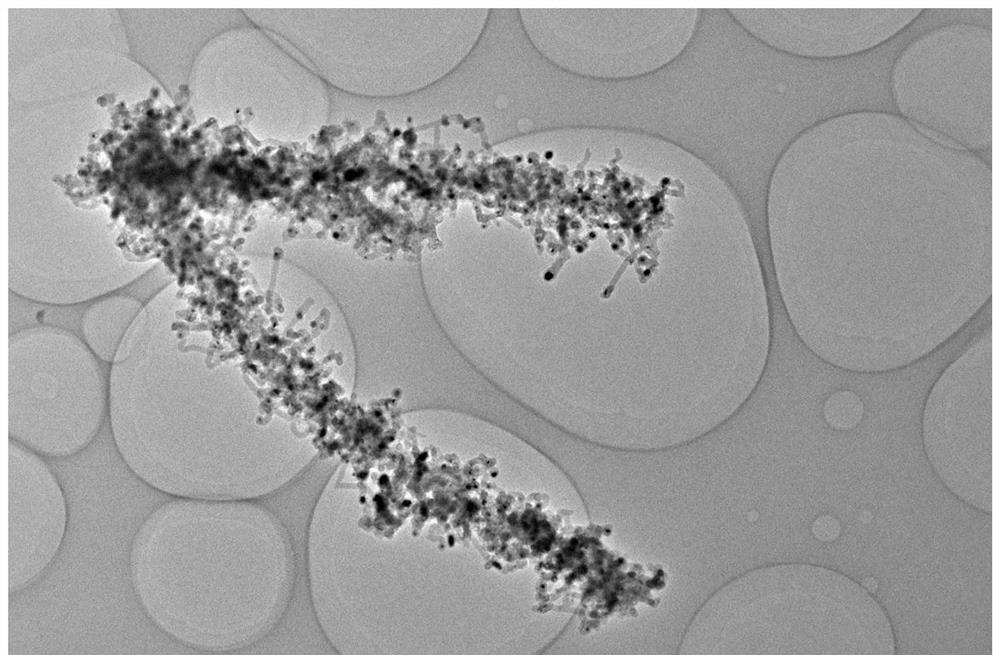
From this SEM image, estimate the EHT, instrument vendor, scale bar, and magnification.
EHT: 200 kV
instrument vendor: FEI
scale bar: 2000 nm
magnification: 7 K X